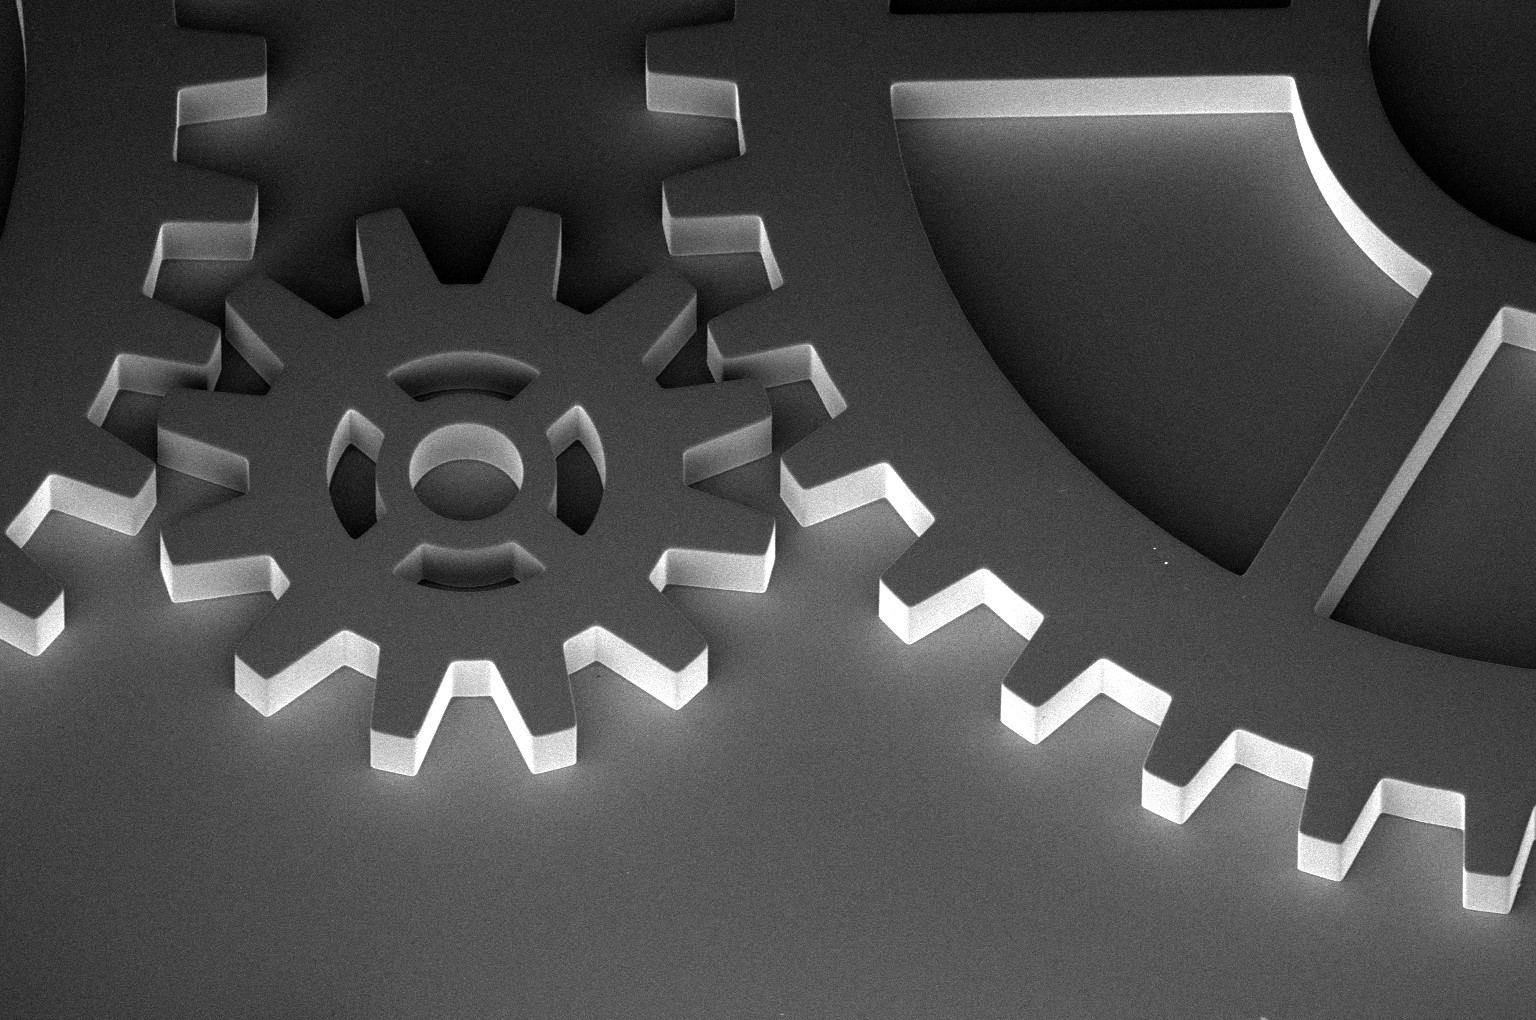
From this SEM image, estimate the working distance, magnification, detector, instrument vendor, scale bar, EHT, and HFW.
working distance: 4 mm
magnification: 0.2 K X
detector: TLD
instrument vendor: FEI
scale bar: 200000 nm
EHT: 2 kV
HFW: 1040 µm